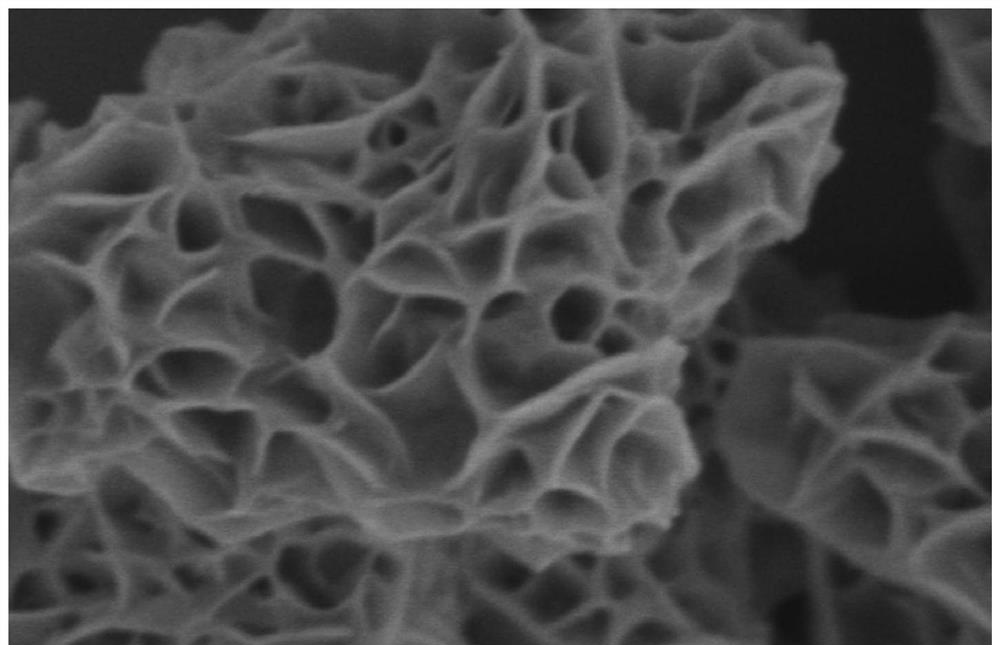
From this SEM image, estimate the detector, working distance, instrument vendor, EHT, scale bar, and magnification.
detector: SE(M)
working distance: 10.8 mm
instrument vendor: Hitachi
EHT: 2 kV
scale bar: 1000 nm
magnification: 50 K X